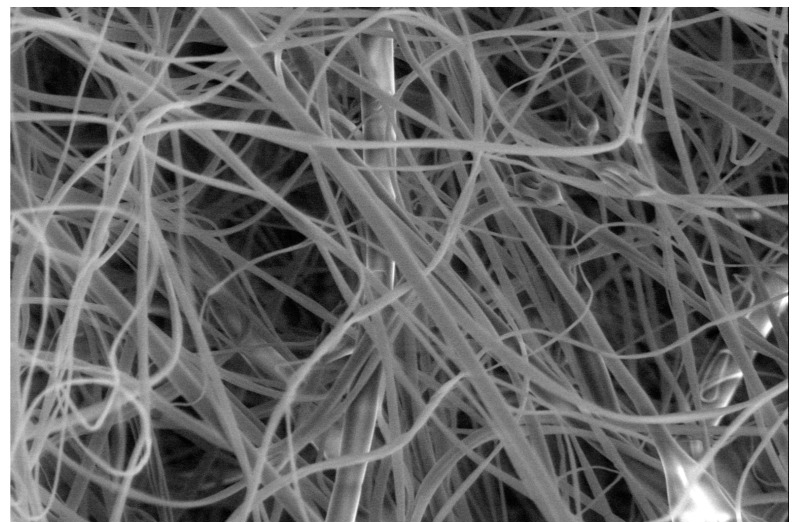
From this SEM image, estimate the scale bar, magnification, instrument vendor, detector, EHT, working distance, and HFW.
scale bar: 10000 nm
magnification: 10 K X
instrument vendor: FEI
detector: LFD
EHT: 5 kV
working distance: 10.3 mm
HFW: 41.4 µm